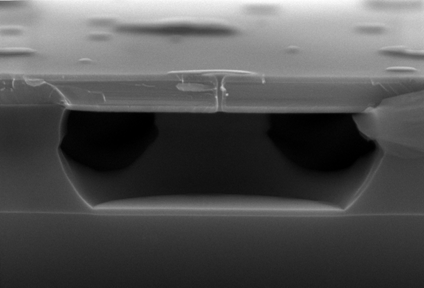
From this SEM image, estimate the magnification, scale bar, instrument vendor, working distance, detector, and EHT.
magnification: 30 K X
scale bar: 1000 nm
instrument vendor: Hitachi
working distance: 6.4 mm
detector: SE(U)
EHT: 15 kV